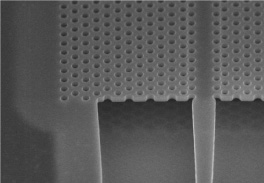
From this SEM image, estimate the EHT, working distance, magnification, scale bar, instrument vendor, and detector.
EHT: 5 kV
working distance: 5 mm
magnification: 15 K X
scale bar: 3000 nm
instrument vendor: Hitachi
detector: SE(M)